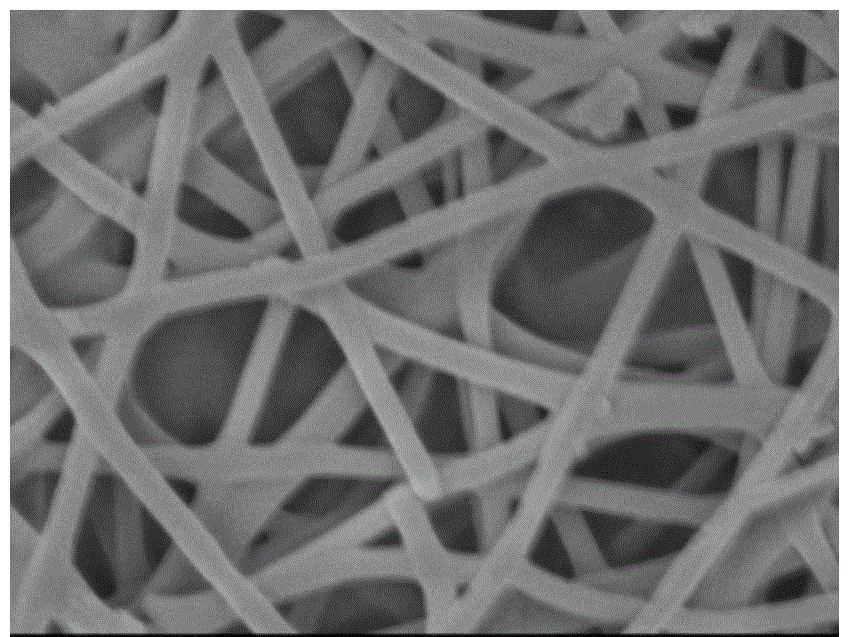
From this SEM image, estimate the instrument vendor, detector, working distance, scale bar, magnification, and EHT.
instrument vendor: JEOL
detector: SEI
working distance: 6.9 mm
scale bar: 100 nm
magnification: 30 K X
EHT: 3 kV